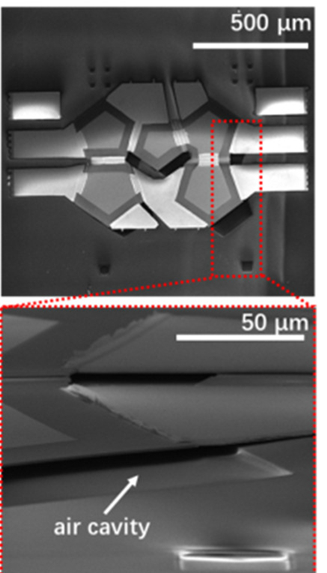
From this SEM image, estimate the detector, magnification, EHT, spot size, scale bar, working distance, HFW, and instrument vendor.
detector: ETD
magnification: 2.4 K X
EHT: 10 kV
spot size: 2.5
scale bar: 50000 nm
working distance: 14 mm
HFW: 124 µm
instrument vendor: FEI